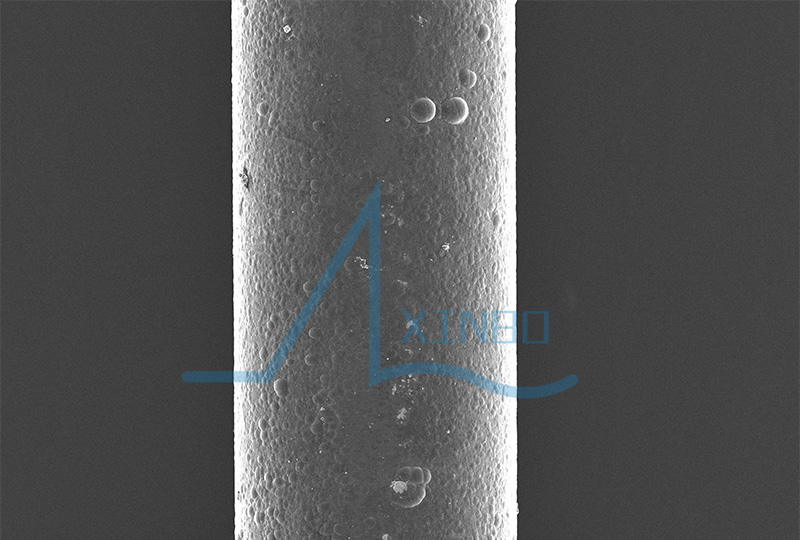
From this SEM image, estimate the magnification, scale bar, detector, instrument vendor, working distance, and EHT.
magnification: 0.1 K X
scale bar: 100000 nm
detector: SED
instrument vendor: JEOL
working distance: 20 mm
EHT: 10 kV